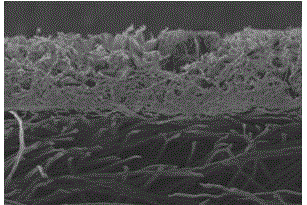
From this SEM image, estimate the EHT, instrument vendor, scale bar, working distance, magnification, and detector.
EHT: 15 kV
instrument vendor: FEI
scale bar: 200000 nm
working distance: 15.5 mm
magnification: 0.1 K X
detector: SE